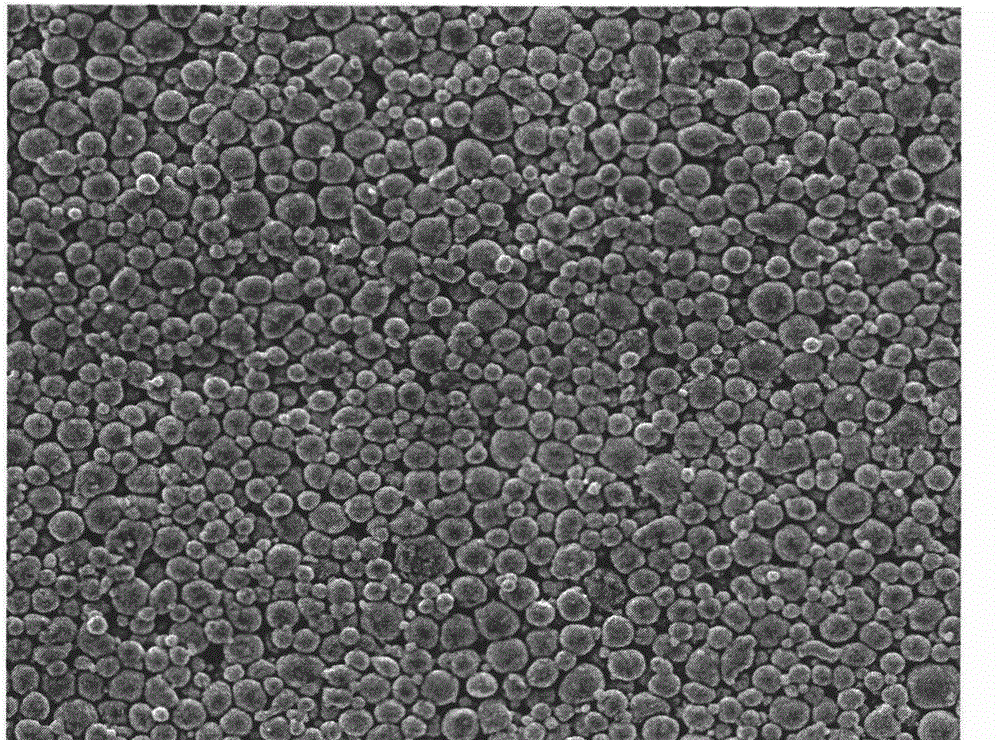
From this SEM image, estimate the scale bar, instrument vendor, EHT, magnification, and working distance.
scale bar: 50000 nm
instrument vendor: FEI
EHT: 20 kV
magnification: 1 K X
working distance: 10 mm